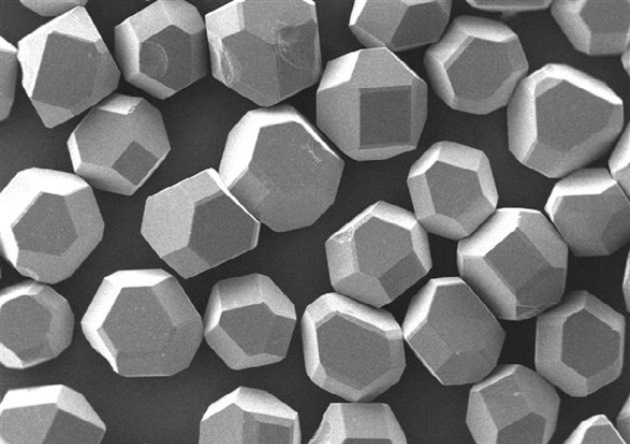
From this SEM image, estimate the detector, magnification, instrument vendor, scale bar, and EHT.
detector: SED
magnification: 0.05 K X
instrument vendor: other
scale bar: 500000 nm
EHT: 20 kV